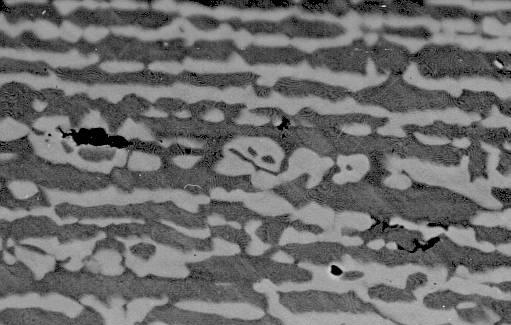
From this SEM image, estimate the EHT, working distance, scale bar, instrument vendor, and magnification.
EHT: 20 kV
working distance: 14 mm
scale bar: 10000 nm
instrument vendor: JEOL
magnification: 2 K X